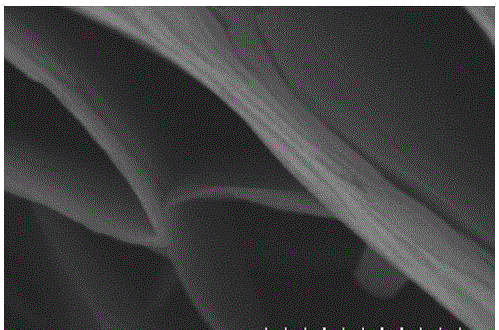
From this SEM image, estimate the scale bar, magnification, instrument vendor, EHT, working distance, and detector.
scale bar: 500 nm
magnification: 100 K X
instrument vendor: Hitachi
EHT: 5 kV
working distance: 7.5 mm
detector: SE(M)